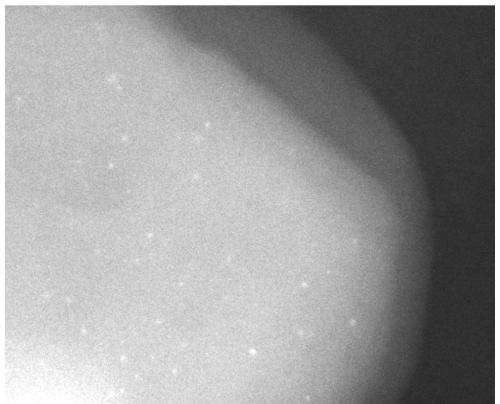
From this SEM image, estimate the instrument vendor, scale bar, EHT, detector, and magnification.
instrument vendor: JEOL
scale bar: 20 nm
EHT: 200 kV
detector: STEM DF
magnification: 1200 K X